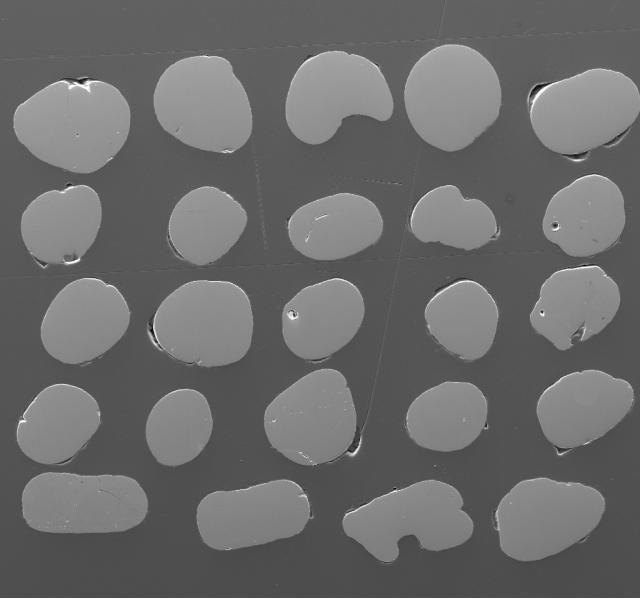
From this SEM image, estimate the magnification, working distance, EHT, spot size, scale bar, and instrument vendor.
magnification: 0.153 K X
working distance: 18.7 mm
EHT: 15 kV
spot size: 6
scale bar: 400000 nm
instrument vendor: FEI